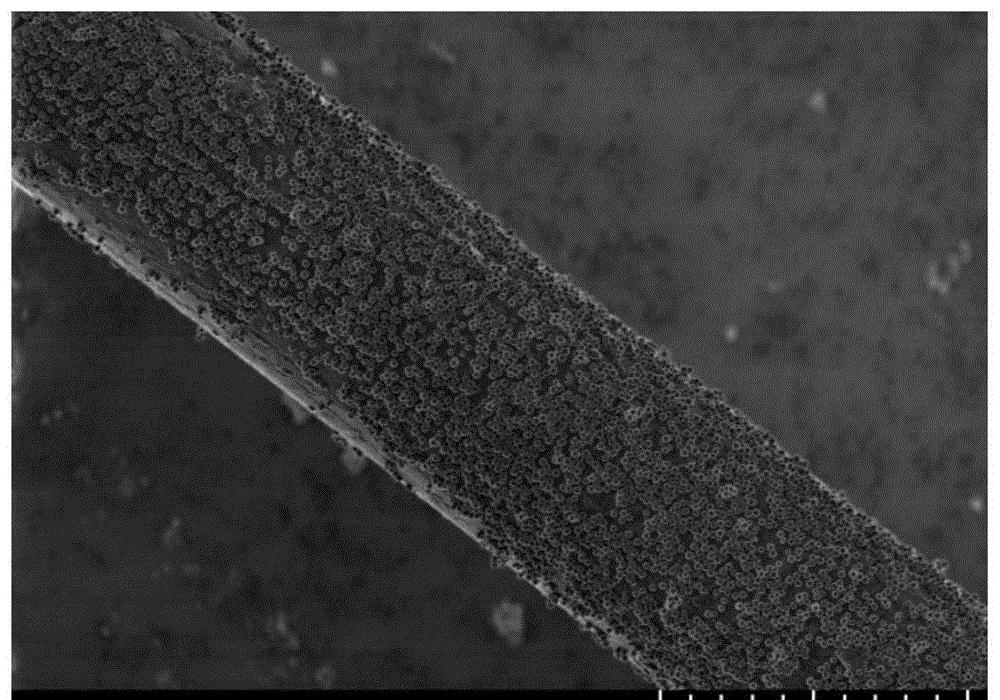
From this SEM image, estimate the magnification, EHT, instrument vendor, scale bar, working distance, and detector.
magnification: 2 K X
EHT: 1 kV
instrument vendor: Hitachi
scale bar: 20000 nm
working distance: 5.1 mm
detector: SE(UL)